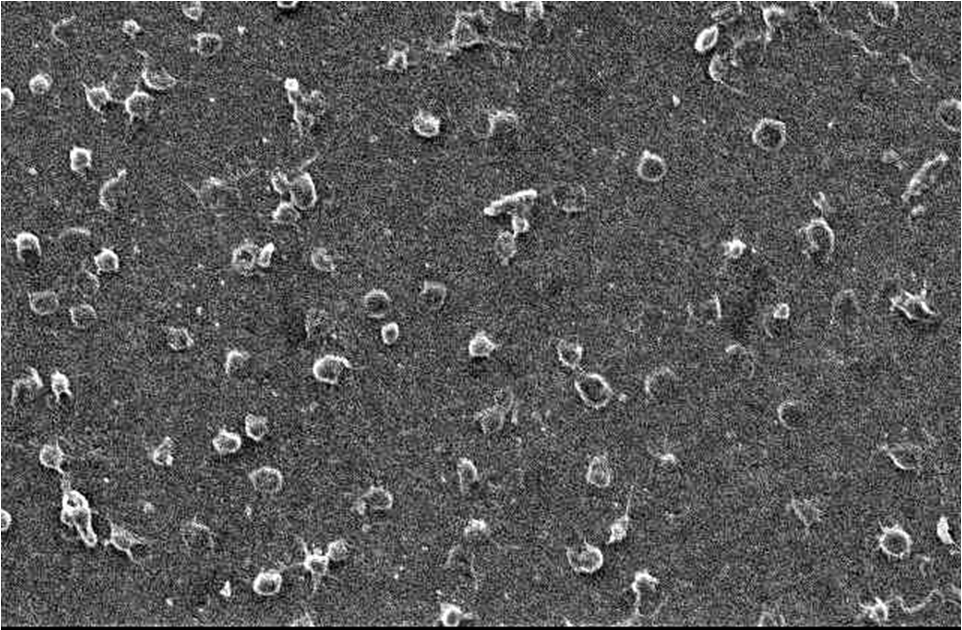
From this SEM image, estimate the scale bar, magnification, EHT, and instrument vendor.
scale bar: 10000 nm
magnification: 1.5 K X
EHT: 5 kV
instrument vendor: JEOL